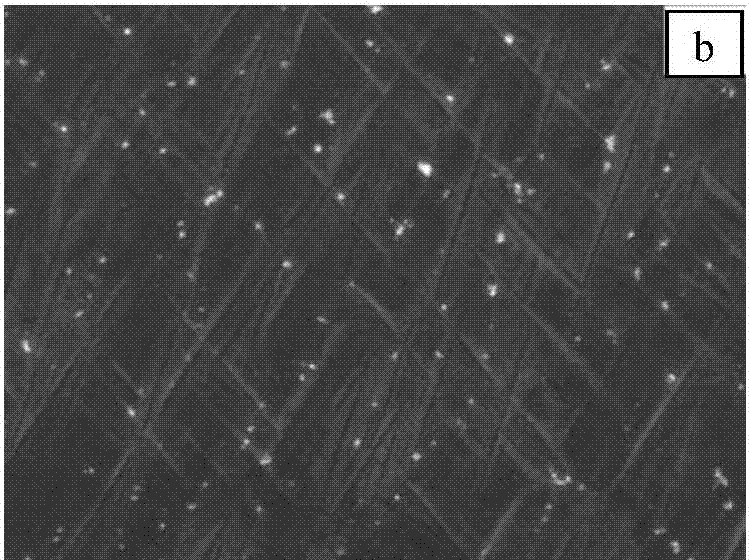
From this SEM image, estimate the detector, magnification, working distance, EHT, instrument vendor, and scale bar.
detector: SEI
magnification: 10 K X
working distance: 8.4 mm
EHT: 5 kV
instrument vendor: JEOL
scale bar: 1000 nm